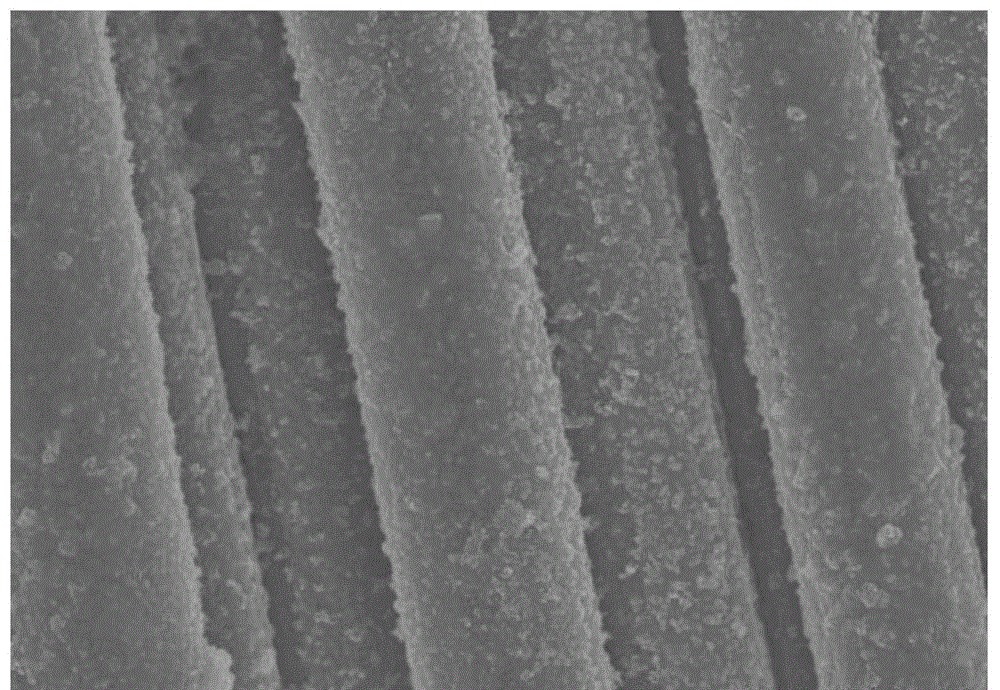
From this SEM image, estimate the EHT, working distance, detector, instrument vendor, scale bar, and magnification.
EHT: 15 kV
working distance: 12.2 mm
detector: SE(M)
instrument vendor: Hitachi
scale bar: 10000 nm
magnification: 3 K X